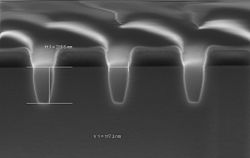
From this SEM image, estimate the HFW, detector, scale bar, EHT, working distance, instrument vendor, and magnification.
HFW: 1.501 µm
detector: InLens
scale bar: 100 nm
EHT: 2 kV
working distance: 2.3 mm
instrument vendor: Zeiss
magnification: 200 K X